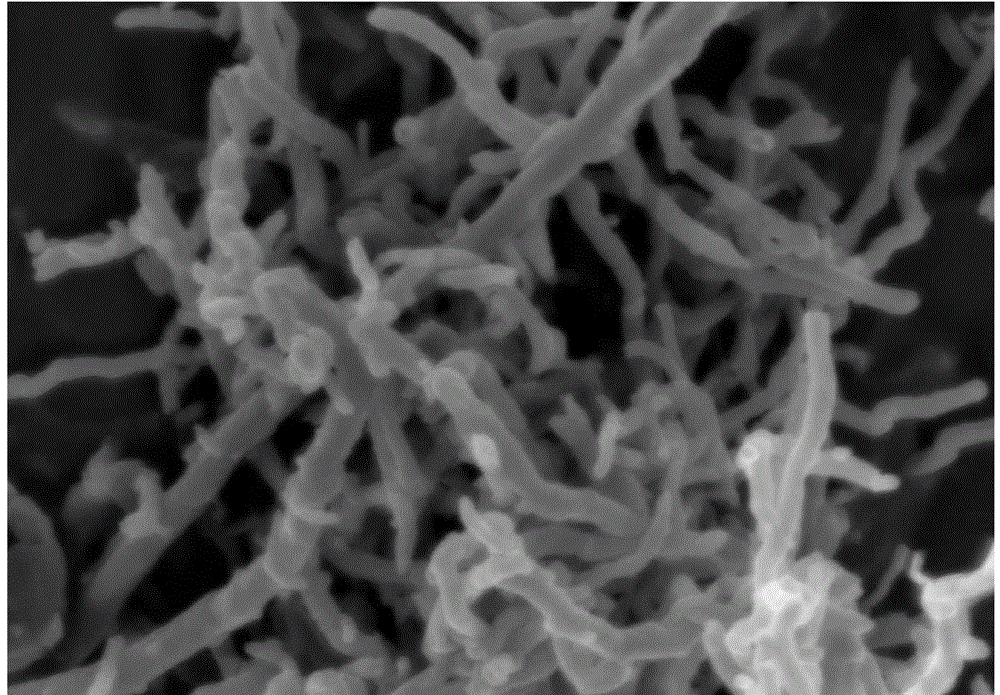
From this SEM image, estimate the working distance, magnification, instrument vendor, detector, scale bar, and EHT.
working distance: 5.2 mm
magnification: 50 K X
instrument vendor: Zeiss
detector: InLens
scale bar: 100 nm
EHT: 3 kV